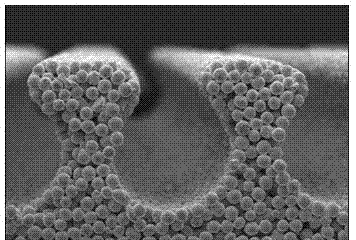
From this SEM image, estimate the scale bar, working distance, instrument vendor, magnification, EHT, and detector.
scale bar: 1000000 nm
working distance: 18.4 mm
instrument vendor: Hitachi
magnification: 0.05 K X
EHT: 10 kV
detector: SE